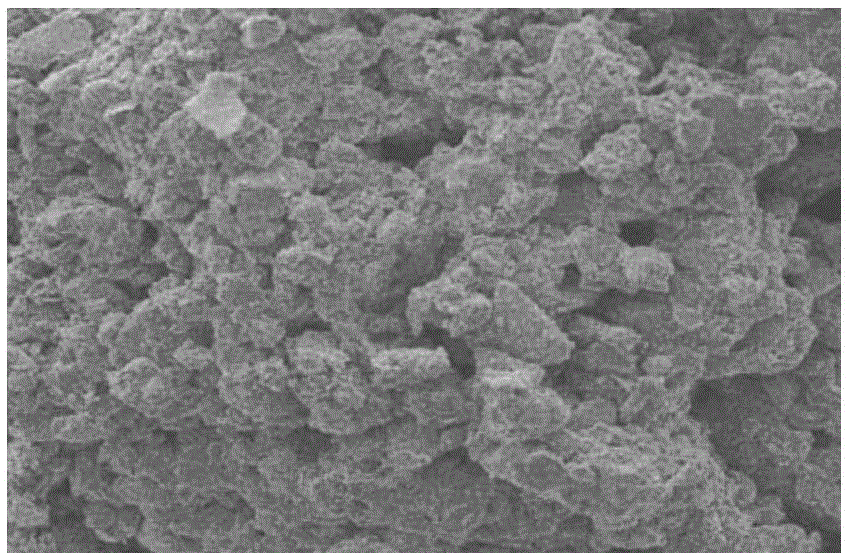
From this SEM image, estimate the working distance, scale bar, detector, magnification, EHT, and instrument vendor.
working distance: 12.3 mm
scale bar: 100000 nm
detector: ETD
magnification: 0.3 K X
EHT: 20 kV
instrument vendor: FEI